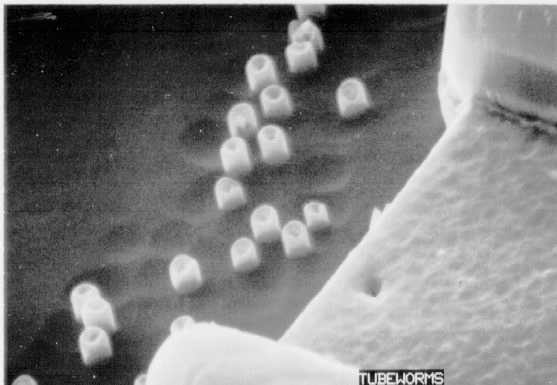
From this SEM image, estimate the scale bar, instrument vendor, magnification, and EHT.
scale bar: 1000 nm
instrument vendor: other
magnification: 15 K X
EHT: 20 kV